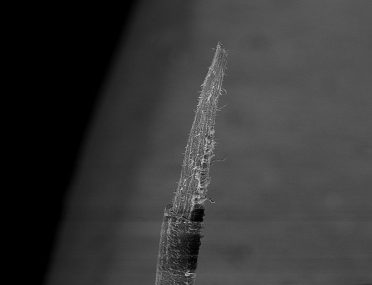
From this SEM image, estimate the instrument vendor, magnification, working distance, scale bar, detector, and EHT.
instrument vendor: Zeiss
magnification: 0.25 K X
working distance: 27.5 mm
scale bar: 100000 nm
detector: SE1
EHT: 10 kV